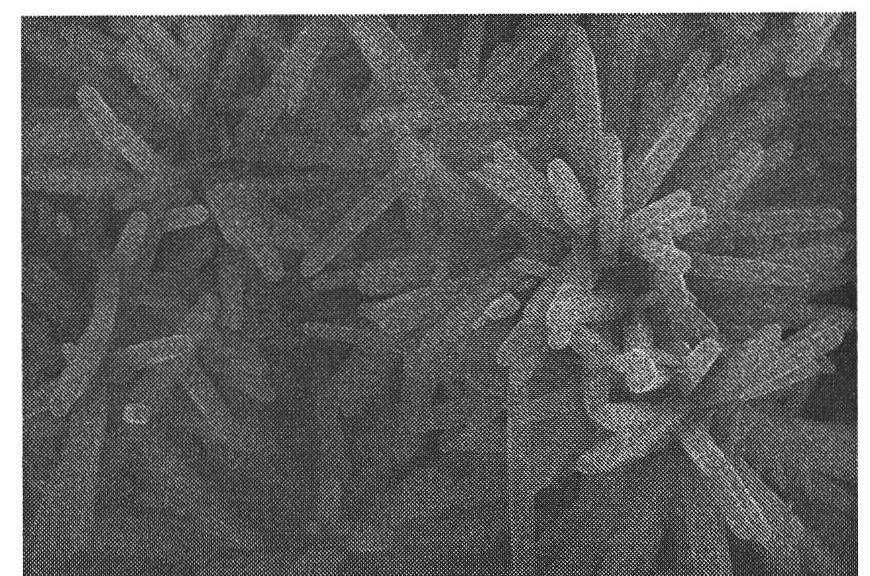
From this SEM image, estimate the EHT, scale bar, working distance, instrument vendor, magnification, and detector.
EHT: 15 kV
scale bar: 5000 nm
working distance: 7 mm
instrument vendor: Hitachi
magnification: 10 K X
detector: SE